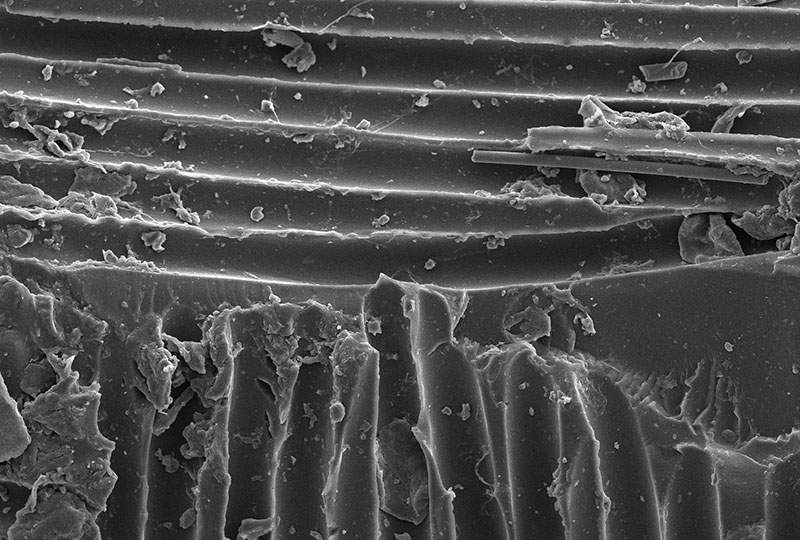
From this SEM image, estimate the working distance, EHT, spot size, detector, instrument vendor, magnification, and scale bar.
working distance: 10 mm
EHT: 10 kV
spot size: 30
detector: SEI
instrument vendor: JEOL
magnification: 0.5 K X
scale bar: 50000 nm